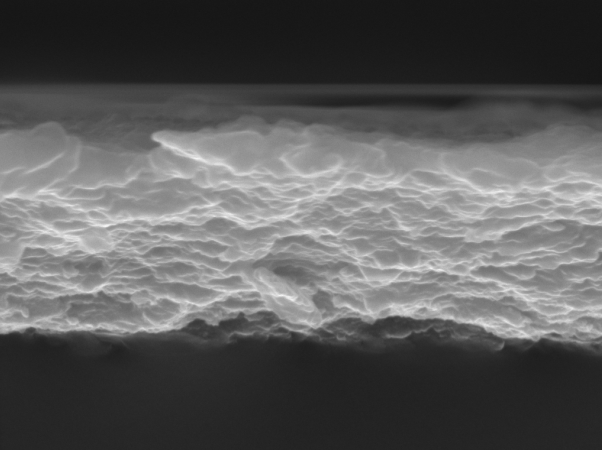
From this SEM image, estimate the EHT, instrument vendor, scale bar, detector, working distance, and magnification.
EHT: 15 kV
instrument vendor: Tescan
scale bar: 1000 nm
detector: SE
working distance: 7.99 mm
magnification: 50 K X